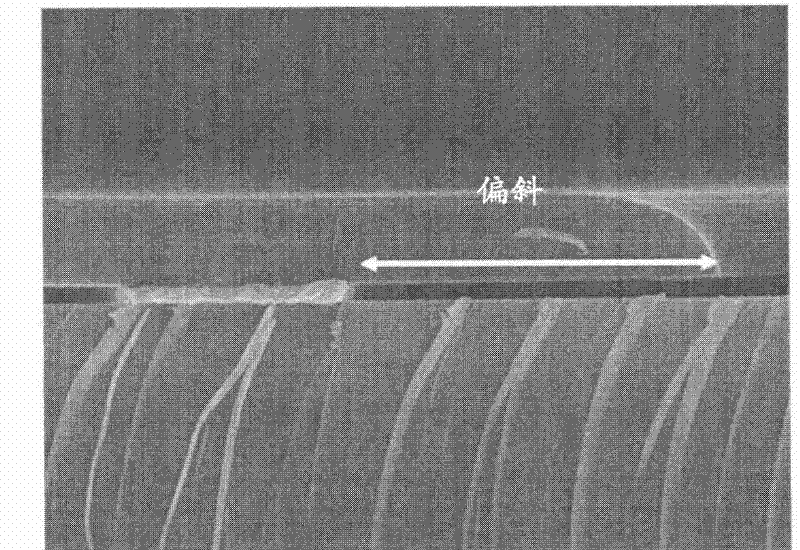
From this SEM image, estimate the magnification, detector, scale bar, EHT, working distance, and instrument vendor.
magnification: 10 K X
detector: SEI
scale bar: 1000 nm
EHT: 15 kV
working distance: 7.8 mm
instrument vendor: JEOL